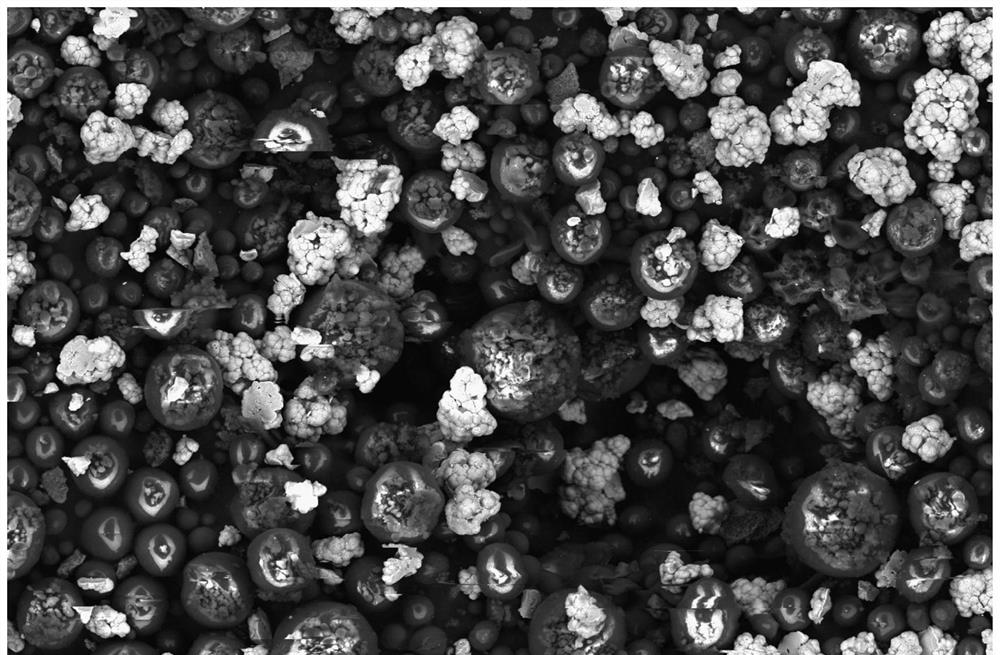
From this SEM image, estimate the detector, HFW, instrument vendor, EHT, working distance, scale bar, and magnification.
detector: CBS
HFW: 423 µm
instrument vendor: Thermo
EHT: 15 kV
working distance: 8.1 mm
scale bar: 100000 nm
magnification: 0.3 K X